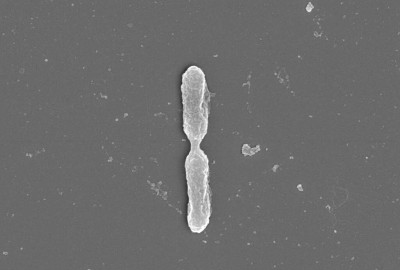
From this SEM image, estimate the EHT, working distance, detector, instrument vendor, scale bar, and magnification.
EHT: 5 kV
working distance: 3.4 mm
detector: InLens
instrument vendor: Zeiss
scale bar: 200 nm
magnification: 20 K X